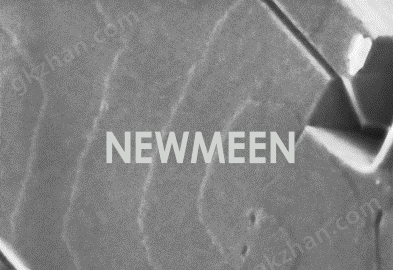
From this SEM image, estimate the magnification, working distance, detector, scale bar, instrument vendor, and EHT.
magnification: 200 K X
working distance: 2.1 mm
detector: UD+TD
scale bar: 200 nm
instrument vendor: Hitachi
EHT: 0.8 kV